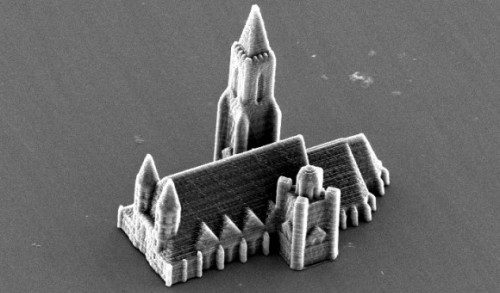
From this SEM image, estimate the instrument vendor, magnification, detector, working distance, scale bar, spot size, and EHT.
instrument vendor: FEI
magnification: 0.817 K X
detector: SE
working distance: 26.8 mm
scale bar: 20000 nm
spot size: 4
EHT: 12 kV